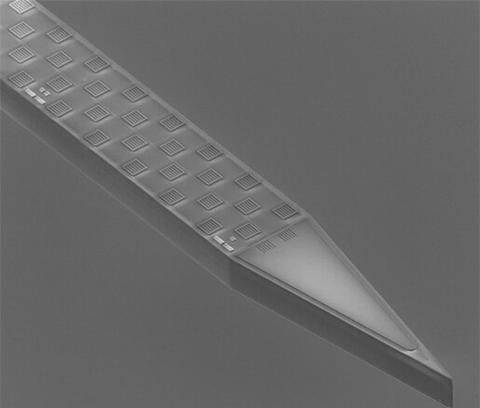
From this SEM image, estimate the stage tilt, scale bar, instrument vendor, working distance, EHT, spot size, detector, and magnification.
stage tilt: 39°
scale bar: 100000 nm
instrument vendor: FEI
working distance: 14.4 mm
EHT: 6.5 kV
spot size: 4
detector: ETD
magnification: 0.8 K X